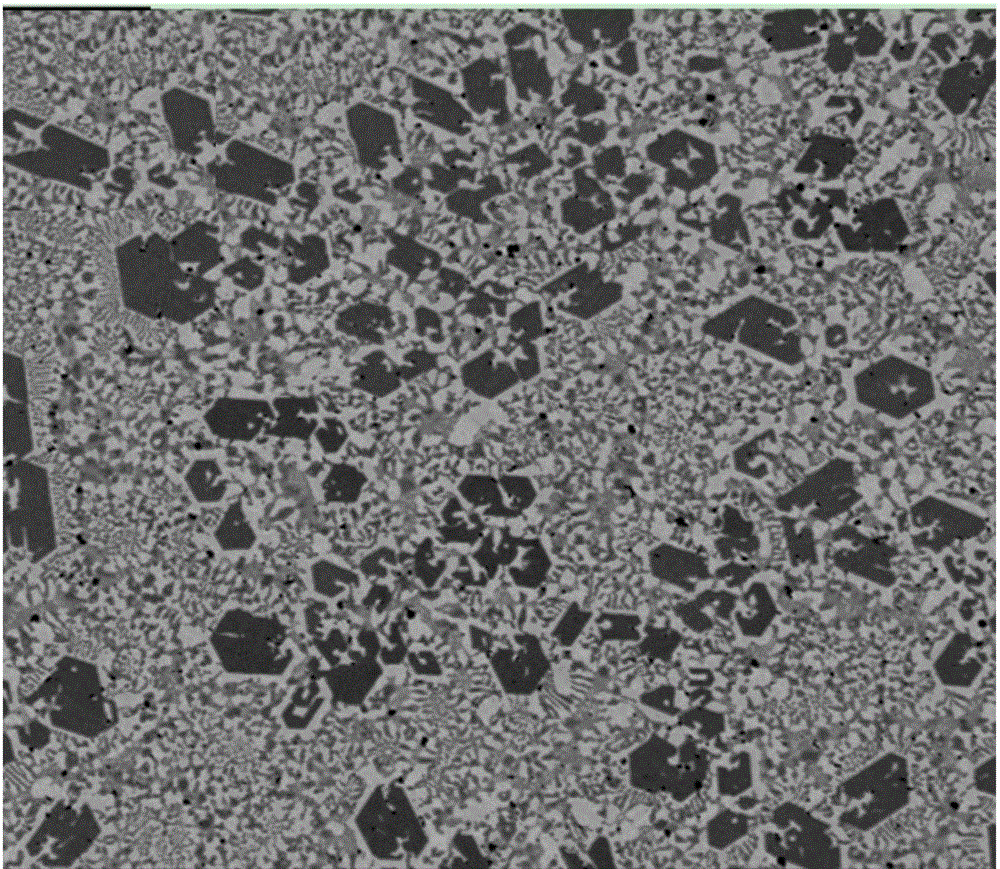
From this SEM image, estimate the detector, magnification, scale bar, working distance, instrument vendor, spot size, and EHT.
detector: SSD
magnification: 0.5 K X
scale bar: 50000 nm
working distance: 10.6 mm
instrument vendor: FEI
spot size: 6.5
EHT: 15 kV